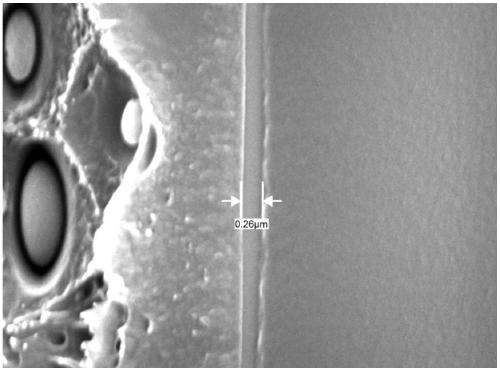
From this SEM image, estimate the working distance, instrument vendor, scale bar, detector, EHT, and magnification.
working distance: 9.5 mm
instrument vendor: JEOL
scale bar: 1000 nm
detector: LED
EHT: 15 kV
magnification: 20 K X